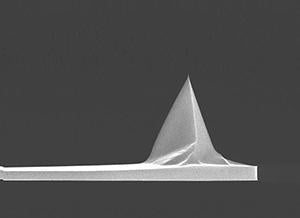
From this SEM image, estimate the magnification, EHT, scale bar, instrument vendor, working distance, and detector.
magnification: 3.5 K X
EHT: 15 kV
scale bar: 10000 nm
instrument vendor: FEI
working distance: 5.6 mm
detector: TLD